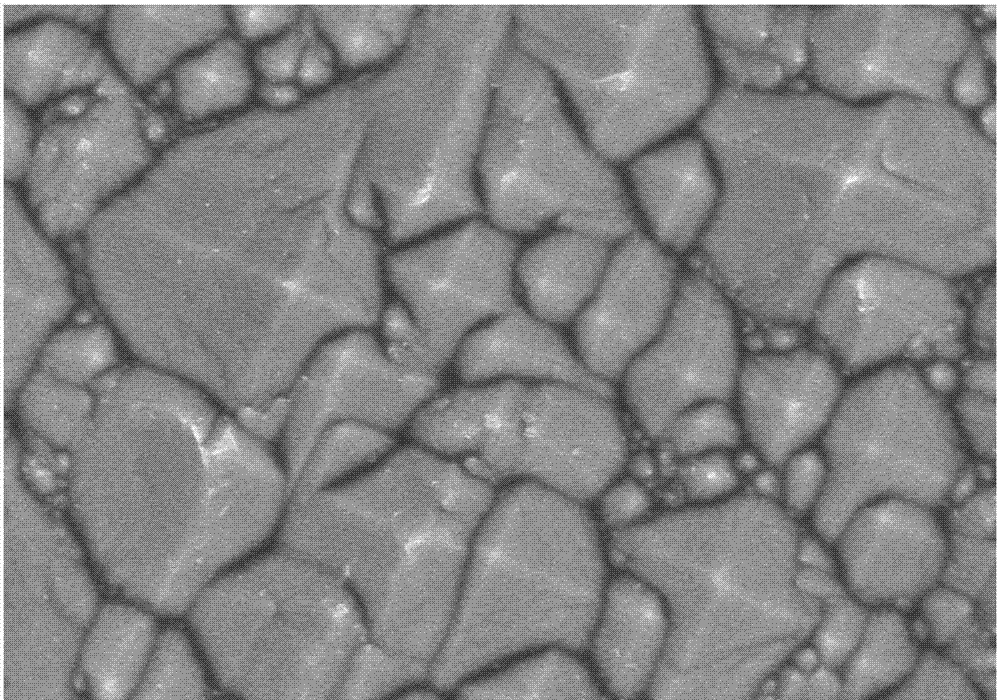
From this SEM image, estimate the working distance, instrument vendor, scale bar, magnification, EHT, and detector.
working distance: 9.2 mm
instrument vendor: Hitachi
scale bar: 5000 nm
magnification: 10 K X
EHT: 6 kV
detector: SE(M)